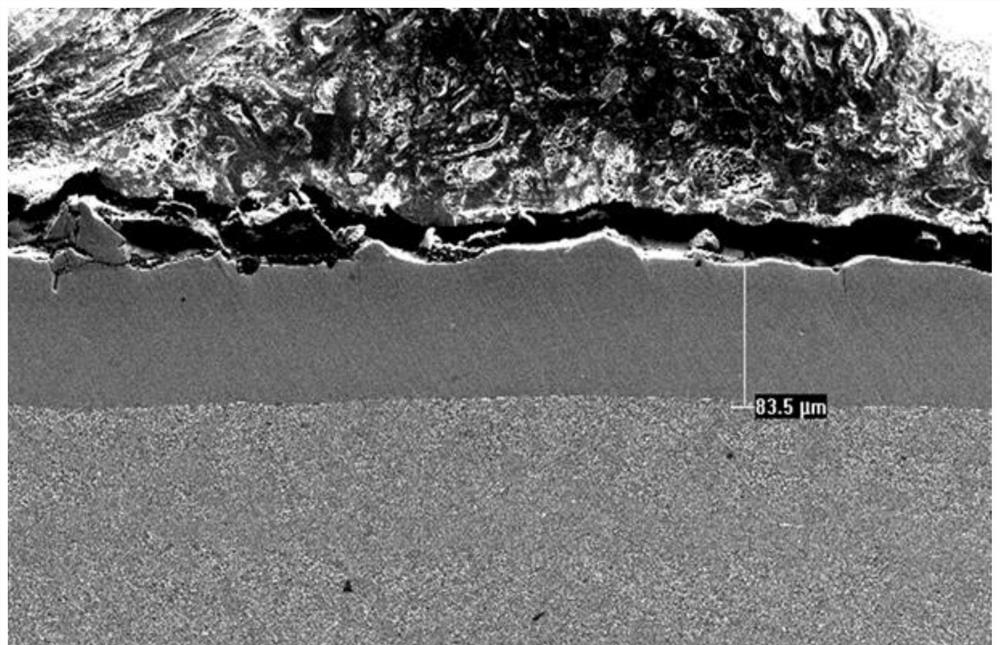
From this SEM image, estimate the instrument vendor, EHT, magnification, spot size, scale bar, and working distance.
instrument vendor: FEI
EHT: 10 kV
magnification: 0.5 K X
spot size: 4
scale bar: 100000 nm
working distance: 8.5 mm